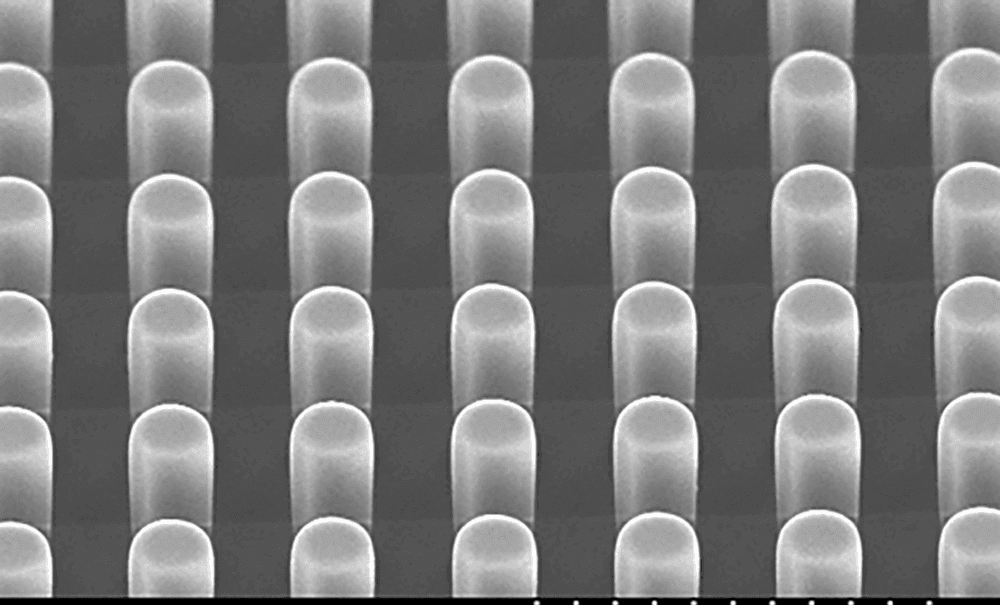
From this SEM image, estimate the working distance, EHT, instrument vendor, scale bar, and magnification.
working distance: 10.1 mm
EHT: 15 kV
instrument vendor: JEOL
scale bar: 100000 nm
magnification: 0.5 K X